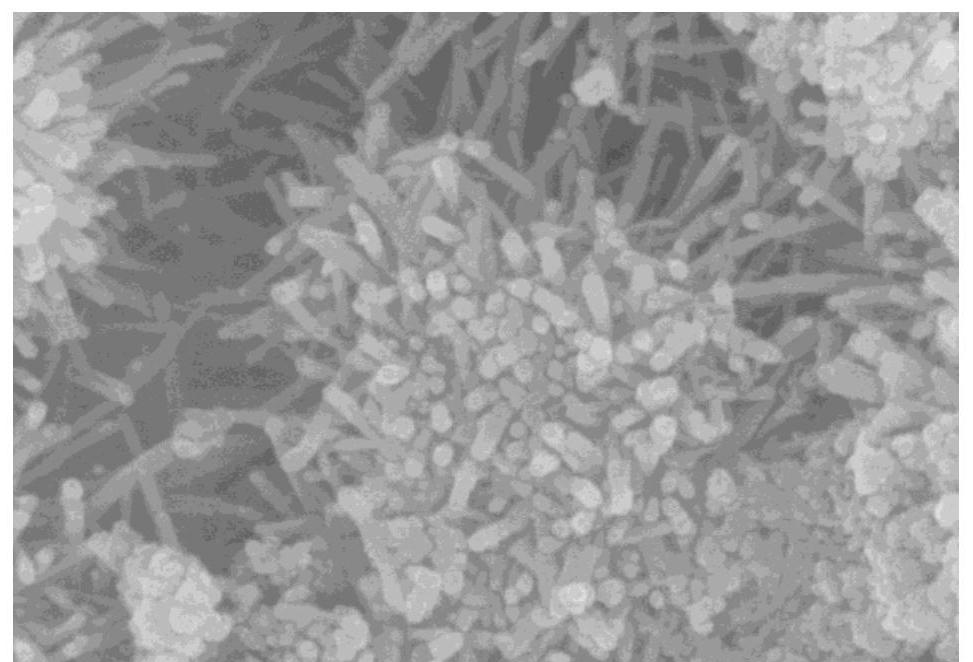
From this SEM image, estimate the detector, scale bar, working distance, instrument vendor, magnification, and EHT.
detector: SE(U)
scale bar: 500 nm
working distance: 9.2 mm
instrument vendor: Hitachi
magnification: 90 K X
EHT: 10 kV